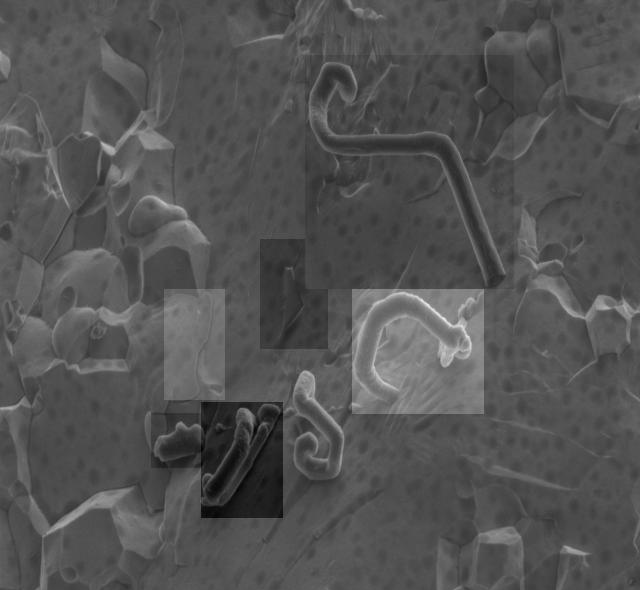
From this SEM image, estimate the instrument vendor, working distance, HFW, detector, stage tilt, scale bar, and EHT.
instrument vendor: FEI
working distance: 4.5 mm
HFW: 20.7 µm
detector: ETD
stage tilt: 0°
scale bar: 5000 nm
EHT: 2 kV